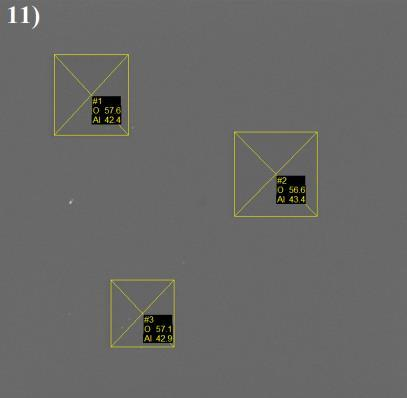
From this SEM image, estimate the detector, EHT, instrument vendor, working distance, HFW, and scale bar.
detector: SE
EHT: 10 kV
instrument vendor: Tescan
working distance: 15.84 mm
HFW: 147 µm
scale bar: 20000 nm